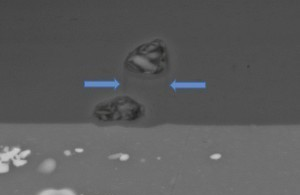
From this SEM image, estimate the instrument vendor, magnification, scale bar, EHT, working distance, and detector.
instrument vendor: Zeiss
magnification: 2.5 K X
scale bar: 2000 nm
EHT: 20 kV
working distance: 23 mm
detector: BSD